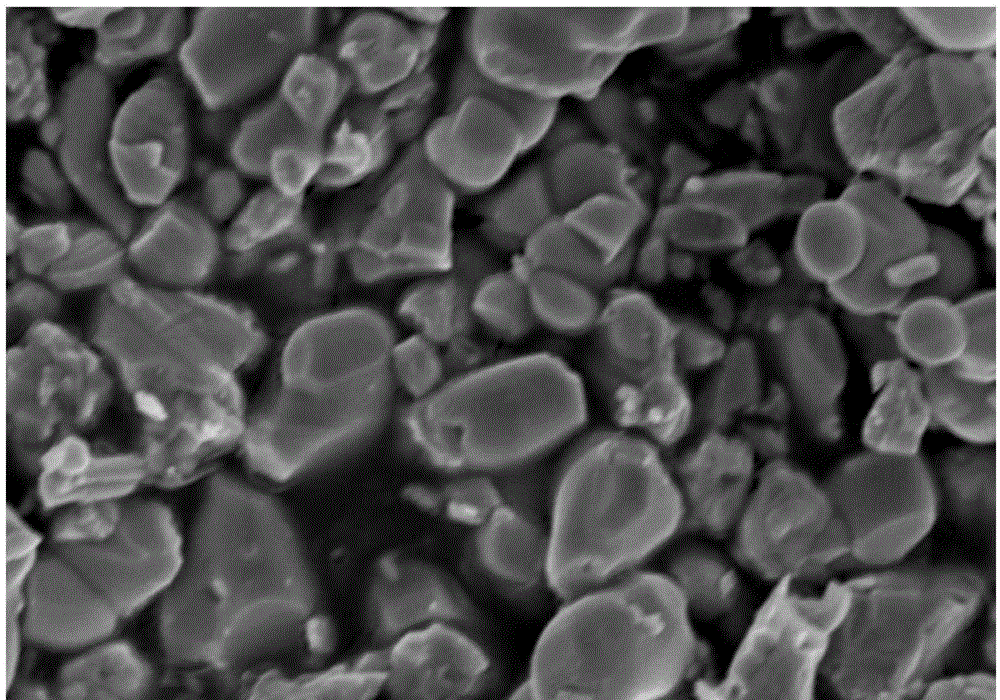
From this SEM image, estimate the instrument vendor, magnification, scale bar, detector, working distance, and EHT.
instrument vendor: Hitachi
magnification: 20 K X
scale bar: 2000 nm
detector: SE(M)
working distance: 8.6 mm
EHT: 5 kV